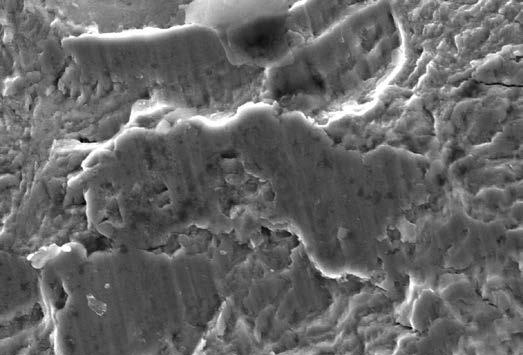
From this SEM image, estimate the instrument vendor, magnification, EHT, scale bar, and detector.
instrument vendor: JEOL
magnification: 2 K X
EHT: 25 kV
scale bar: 10000 nm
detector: SEI